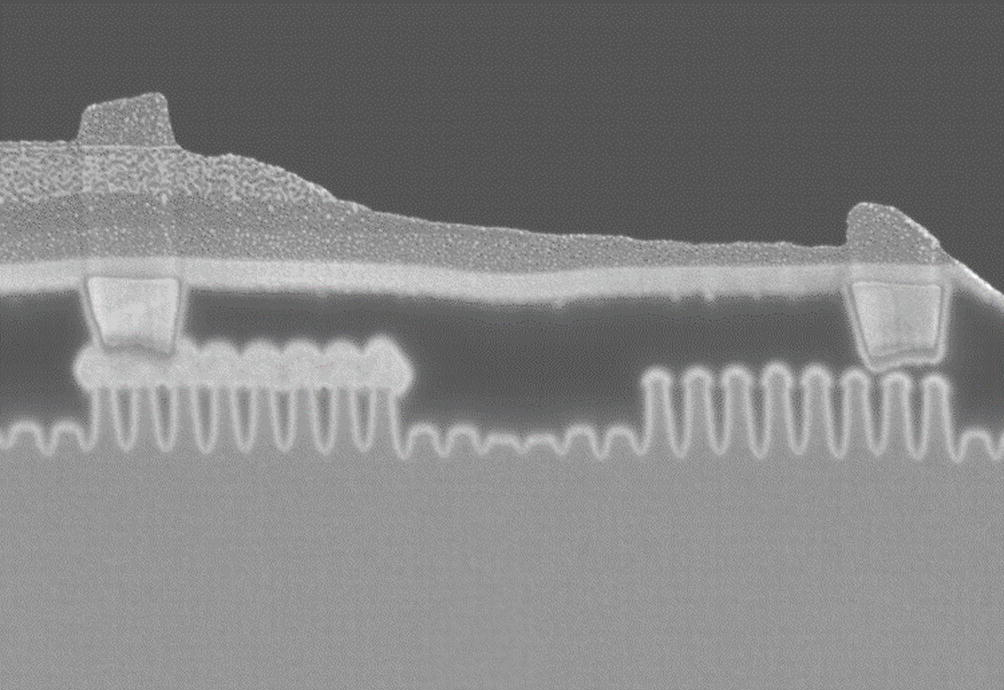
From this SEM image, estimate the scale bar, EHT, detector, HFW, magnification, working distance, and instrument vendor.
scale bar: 100 nm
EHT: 15 kV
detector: InLens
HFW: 1.213 µm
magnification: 94.28 K X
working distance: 1.7 mm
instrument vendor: Zeiss